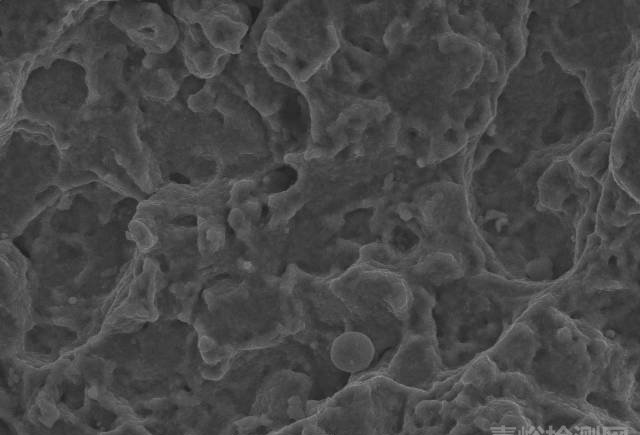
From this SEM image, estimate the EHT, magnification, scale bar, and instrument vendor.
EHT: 20 kV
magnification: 2 K X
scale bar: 10000 nm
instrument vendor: JEOL